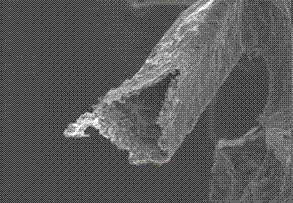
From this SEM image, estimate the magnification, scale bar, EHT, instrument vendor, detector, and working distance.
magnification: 0.5 K X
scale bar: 100000 nm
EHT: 15 kV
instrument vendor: Hitachi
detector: SE(M)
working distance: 14.7 mm